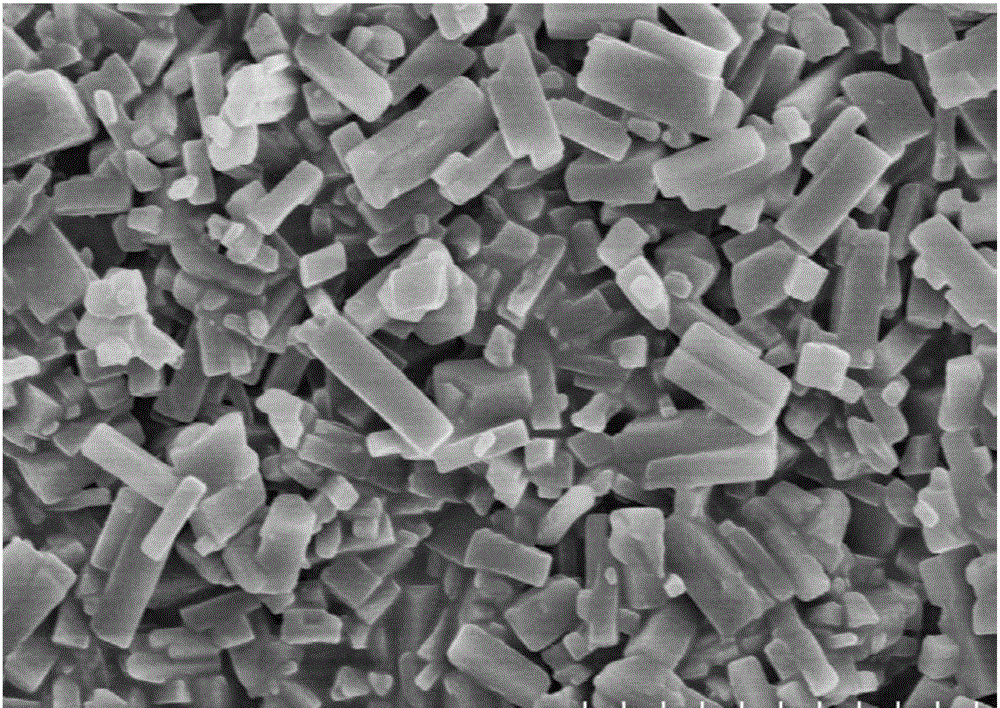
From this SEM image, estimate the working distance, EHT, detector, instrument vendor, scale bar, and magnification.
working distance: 17 mm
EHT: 5 kV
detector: SE(M)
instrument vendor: Hitachi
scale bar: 5000 nm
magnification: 10 K X